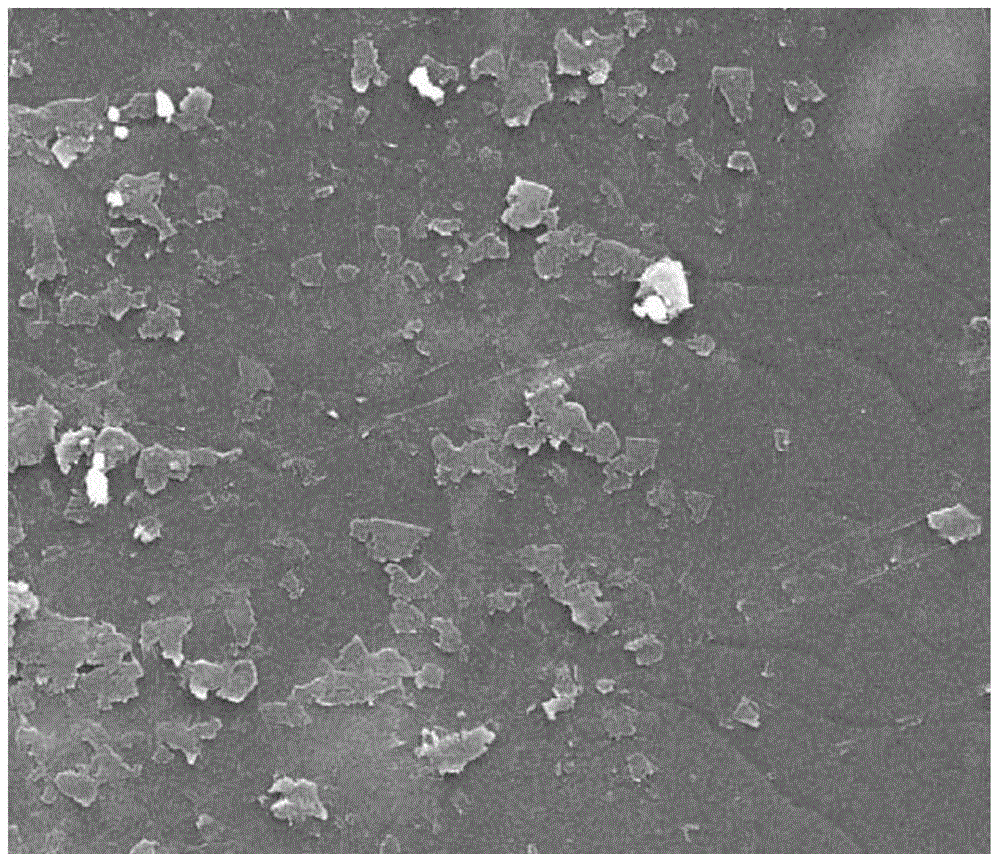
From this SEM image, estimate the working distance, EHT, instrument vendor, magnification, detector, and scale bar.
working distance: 10.7 mm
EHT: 30 kV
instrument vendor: FEI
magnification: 2 K X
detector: SE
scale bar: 50000 nm